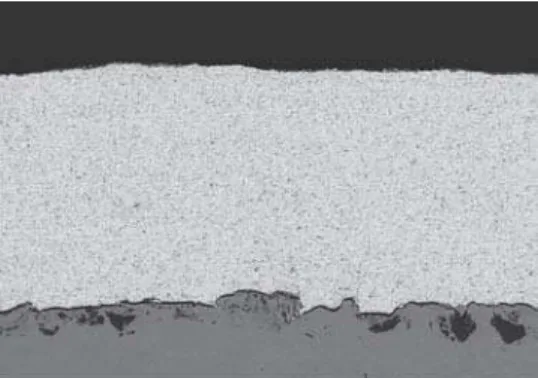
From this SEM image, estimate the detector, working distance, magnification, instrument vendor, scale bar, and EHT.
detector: YAGBSE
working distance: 16.7 mm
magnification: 0.5 K X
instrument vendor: Hitachi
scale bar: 100000 nm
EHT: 15 kV